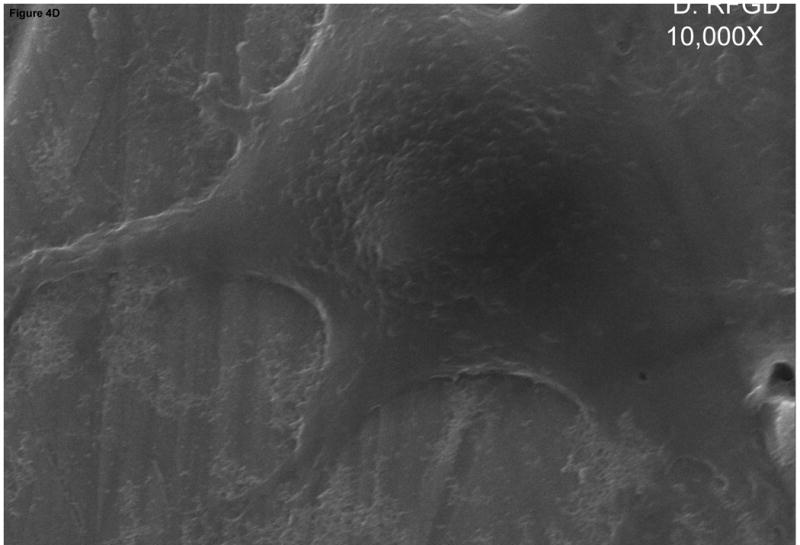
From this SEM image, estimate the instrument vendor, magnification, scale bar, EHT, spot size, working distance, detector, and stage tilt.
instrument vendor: FEI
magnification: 10 K X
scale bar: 5000 nm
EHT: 20 kV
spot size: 3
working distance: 9.97 mm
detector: Etd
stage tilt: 0.2°